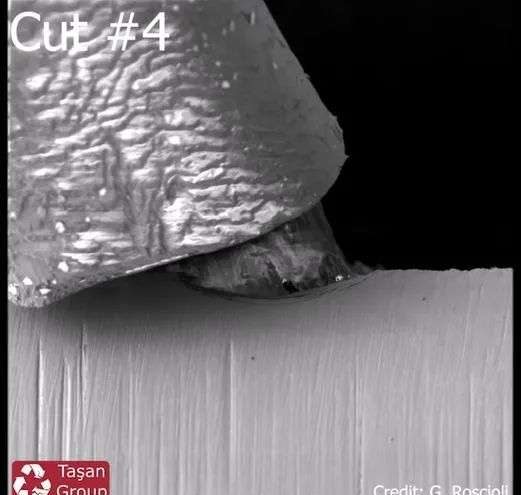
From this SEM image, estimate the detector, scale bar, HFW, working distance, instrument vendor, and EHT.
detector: SE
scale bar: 20000 nm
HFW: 149 µm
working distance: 10.04 mm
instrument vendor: Tescan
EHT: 2 kV